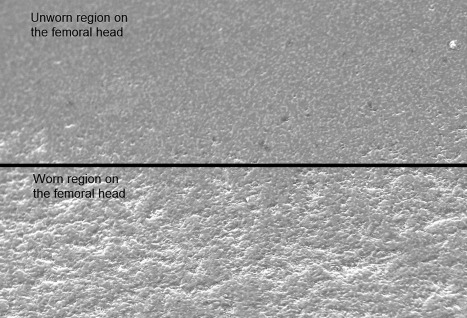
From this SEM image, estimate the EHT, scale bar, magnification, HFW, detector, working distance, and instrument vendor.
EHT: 20 kV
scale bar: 10000 nm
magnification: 3 K X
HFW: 99.02 µm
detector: SE1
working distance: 14 mm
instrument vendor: Zeiss